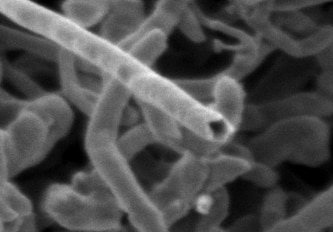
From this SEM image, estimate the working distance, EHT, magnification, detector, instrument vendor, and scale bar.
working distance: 2.9 mm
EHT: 0.5 kV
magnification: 200 K X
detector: SE(T)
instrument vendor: Hitachi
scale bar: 200 nm